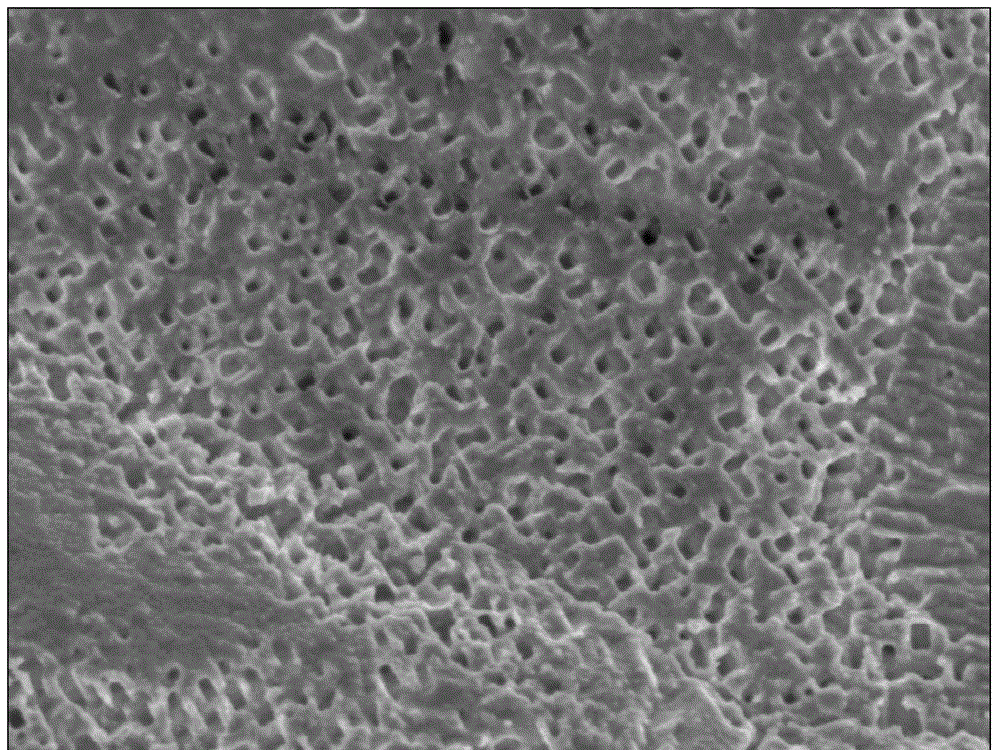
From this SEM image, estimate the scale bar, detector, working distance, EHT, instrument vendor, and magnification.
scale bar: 1000 nm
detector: SEI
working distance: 5.9 mm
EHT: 5 kV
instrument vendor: JEOL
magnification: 15 K X